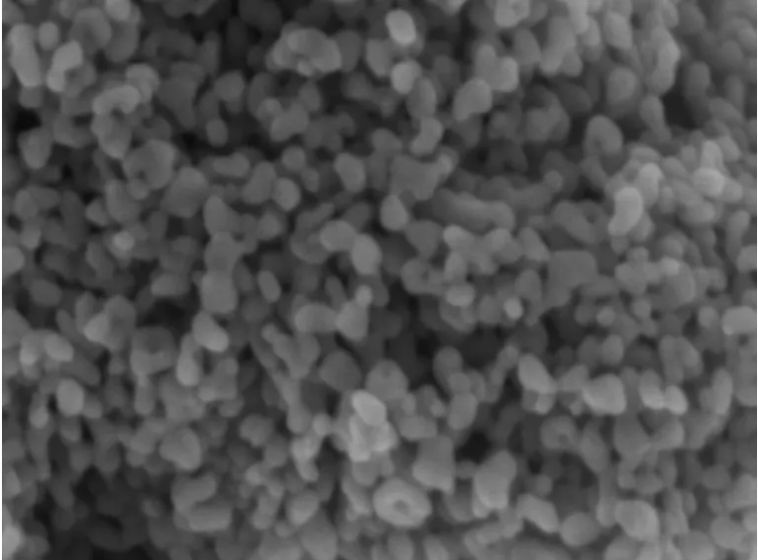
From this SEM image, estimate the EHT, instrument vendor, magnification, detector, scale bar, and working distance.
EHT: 3 kV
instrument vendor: Zeiss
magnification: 200 K X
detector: InLens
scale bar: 100 nm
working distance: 4.8 mm